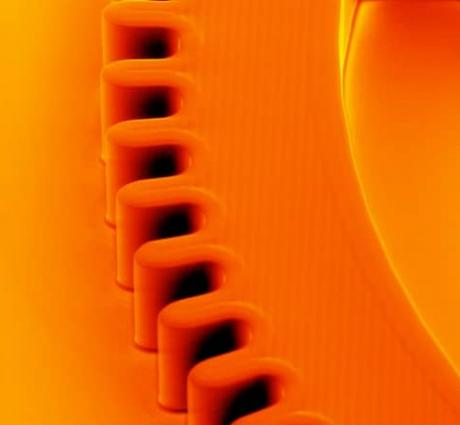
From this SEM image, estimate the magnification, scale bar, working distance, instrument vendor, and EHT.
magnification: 0.253 K X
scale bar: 500000 nm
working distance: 13.3 mm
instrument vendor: FEI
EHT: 15 kV